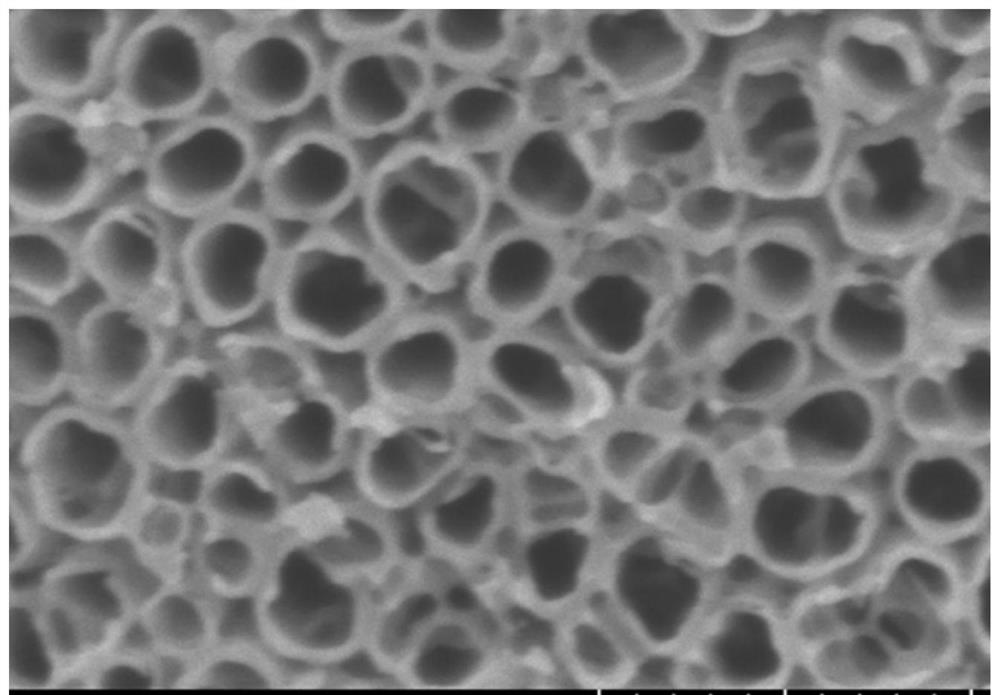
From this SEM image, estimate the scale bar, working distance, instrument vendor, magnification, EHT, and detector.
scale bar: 400 nm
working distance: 8.1 mm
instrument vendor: Hitachi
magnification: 120 K X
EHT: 3 kV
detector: SE(U)